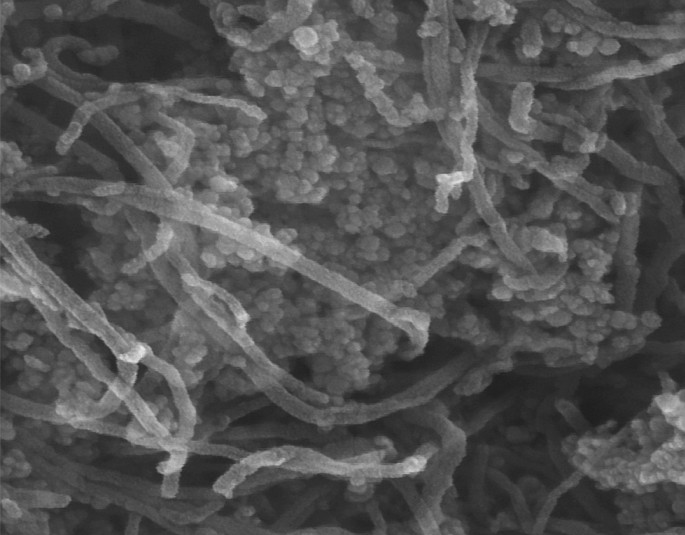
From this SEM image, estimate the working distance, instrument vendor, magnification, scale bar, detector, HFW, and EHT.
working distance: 4.95 mm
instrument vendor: Tescan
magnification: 250 K X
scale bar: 200 nm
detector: In-Beam SE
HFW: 0.83 µm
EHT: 15 kV